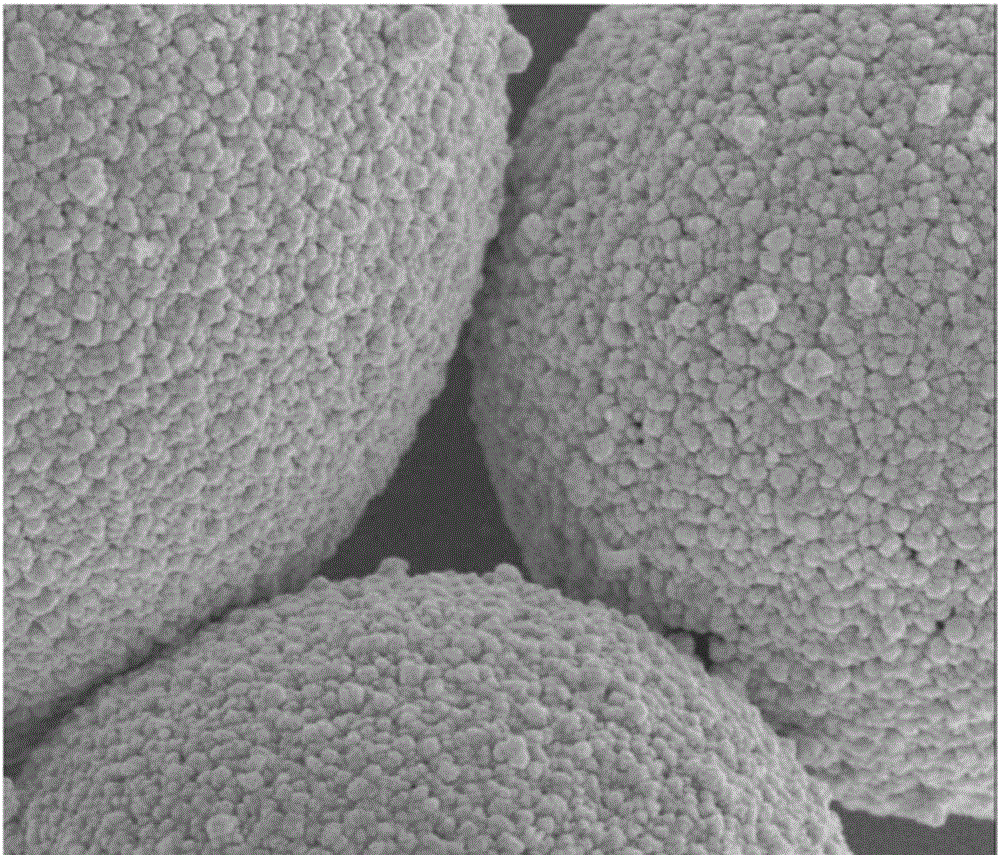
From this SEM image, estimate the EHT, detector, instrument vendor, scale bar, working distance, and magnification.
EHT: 5 kV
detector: TLD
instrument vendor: FEI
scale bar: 1000 nm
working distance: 4.8 mm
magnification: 30 K X